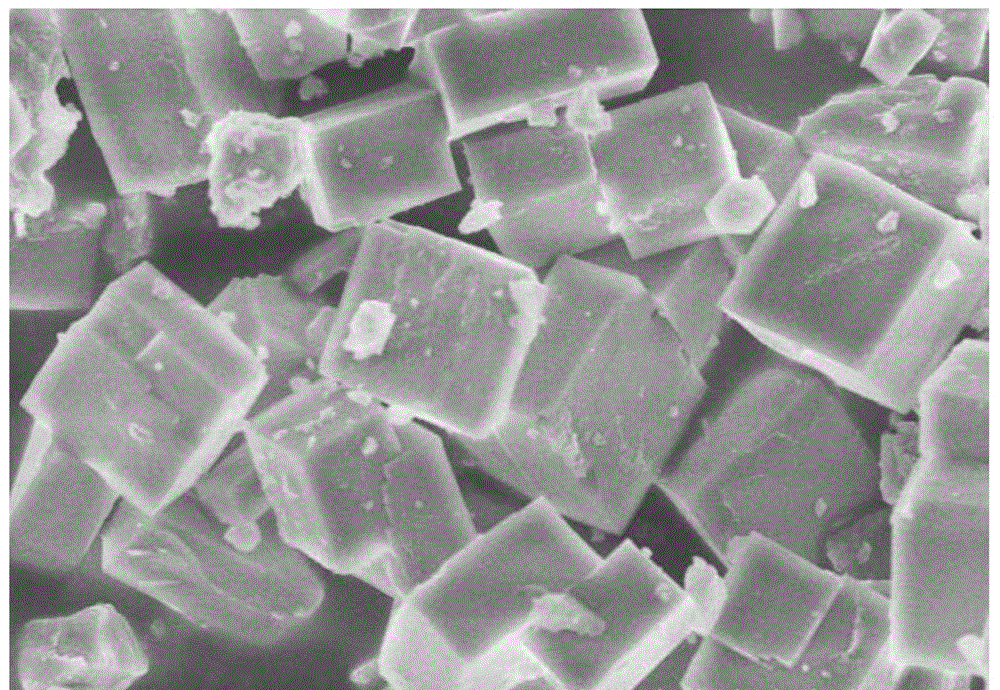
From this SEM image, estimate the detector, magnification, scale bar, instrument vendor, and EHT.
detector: SE(M)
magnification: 10 K X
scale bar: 5000 nm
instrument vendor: Hitachi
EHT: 5 kV